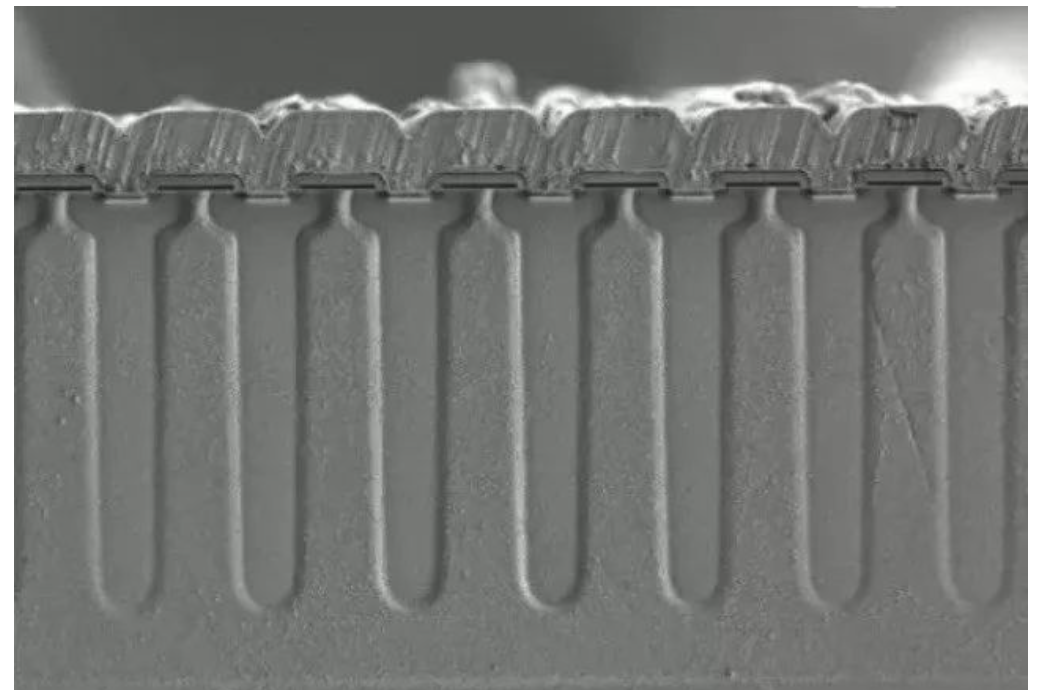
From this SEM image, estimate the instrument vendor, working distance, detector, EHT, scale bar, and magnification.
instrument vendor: Hitachi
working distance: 10.2 mm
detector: SE(L)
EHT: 1 kV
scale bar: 40000 nm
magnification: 1.3 K X